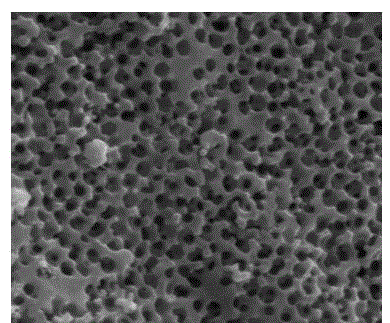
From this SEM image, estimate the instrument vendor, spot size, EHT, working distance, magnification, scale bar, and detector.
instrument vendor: FEI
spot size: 4.5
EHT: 18 kV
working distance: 8.2 mm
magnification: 100 K X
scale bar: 500 nm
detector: ETD